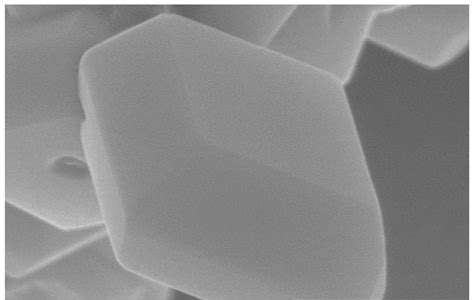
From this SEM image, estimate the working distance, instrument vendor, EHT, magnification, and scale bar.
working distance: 11.6 mm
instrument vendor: Hitachi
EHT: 15 kV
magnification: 45 K X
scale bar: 1000 nm